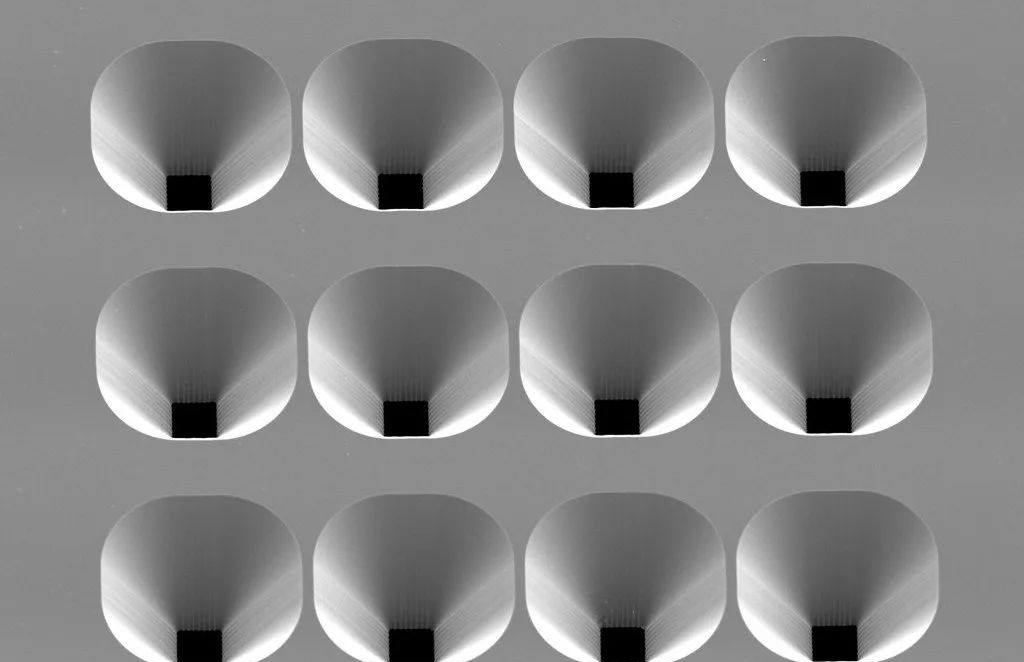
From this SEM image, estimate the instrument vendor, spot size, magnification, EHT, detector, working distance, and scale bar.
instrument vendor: JEOL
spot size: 50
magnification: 0.33 K X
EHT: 10 kV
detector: SEI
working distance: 37 mm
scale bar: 50000 nm